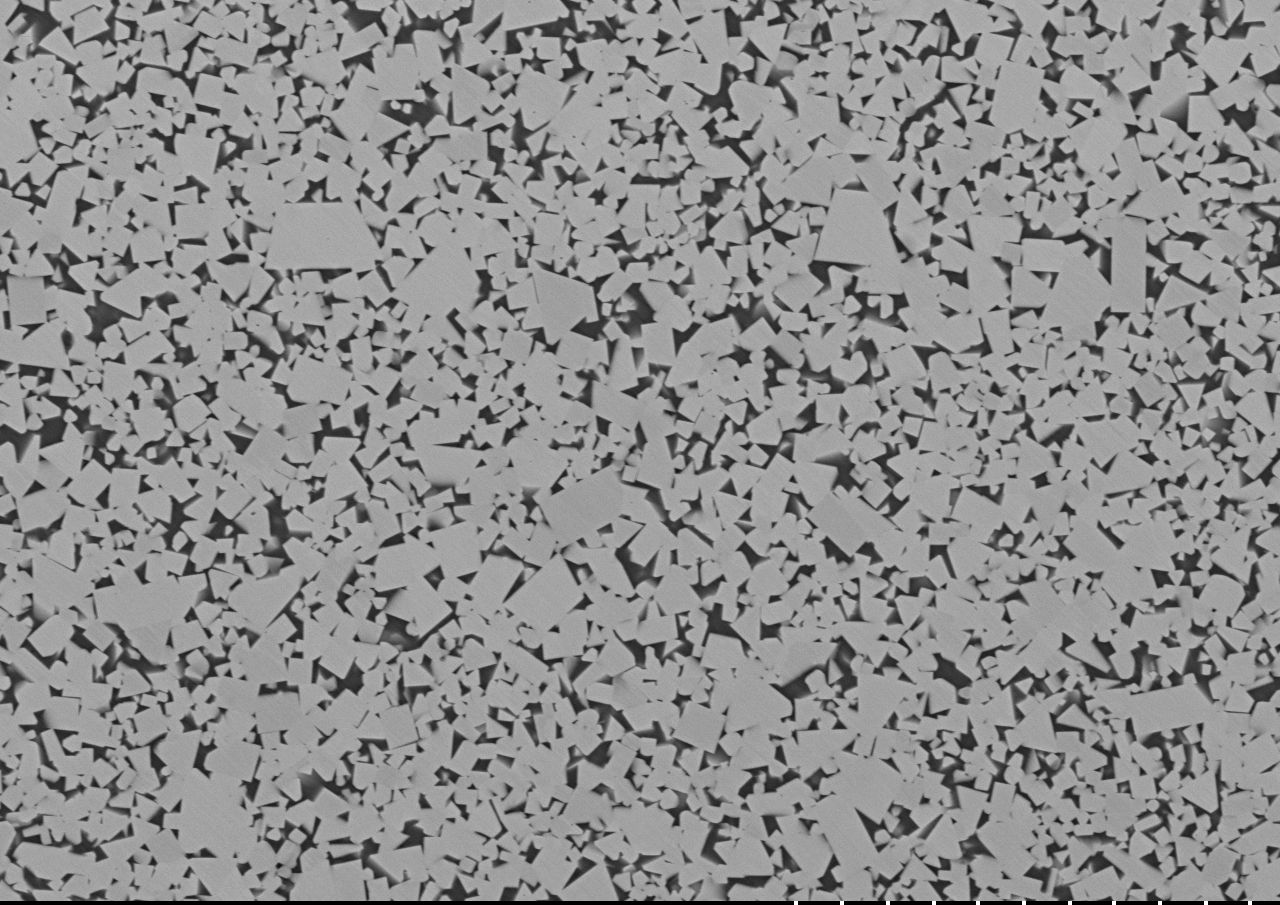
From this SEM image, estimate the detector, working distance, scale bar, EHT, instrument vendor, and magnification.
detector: BSE-COMP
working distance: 5.8 mm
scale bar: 30000 nm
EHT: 20 kV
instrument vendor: Hitachi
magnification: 1.5 K X